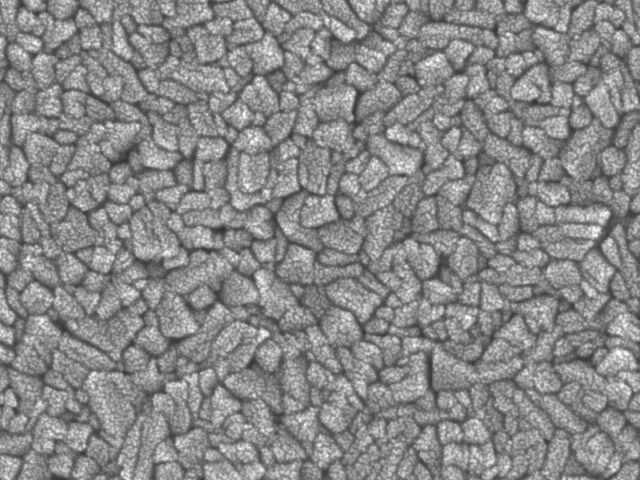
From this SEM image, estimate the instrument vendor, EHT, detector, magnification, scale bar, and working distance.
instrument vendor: JEOL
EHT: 5 kV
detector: SEI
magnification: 200 K X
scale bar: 100 nm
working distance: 2 mm